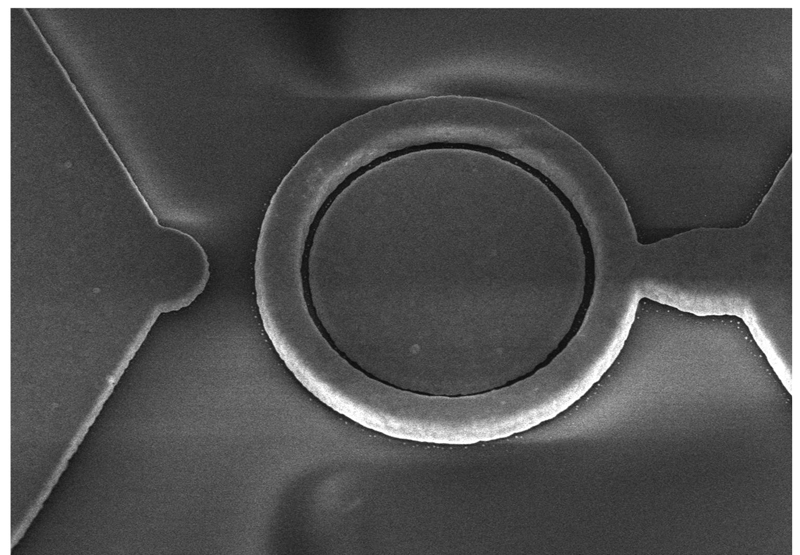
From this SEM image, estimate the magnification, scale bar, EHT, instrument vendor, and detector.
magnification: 15 K X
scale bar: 3000 nm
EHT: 5 kV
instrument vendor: Hitachi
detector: SE(U)